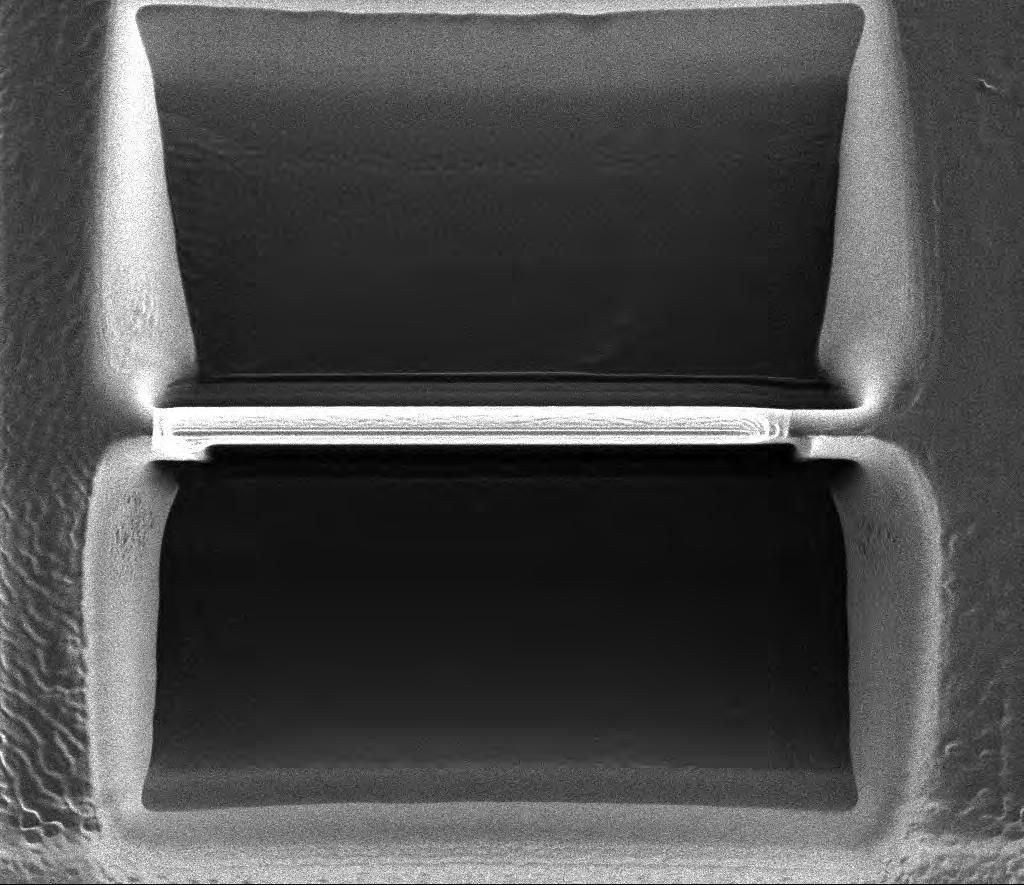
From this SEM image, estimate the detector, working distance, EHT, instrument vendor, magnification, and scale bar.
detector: ETD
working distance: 19 mm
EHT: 30 kV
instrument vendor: FEI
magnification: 5 K X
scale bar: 10000 nm